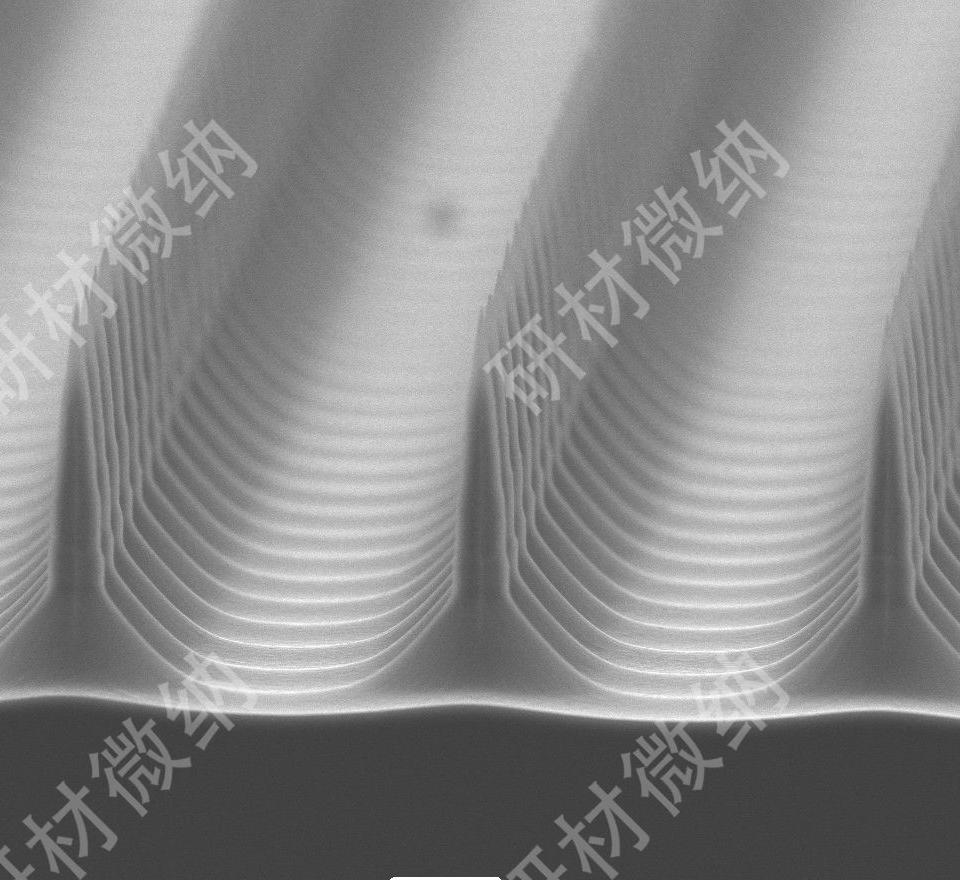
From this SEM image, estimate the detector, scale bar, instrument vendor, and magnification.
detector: SEI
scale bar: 20000 nm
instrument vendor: JEOL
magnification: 0.55 K X